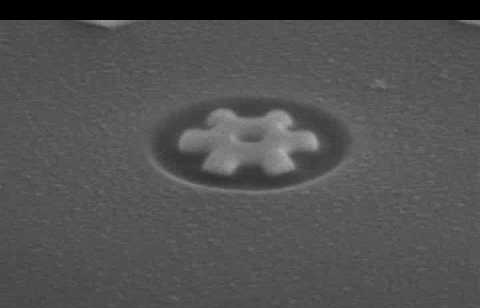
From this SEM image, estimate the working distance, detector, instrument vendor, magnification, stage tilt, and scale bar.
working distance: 5.1 mm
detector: ETD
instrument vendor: FEI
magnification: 130.002 K X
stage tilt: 52°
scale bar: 500 nm